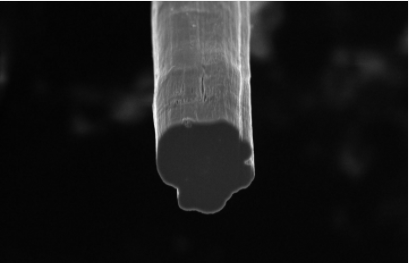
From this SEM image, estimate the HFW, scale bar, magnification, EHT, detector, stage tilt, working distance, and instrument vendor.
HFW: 42.2 µm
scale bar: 5000 nm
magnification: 4.908 K X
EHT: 5 kV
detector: TLD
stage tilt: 20°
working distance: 3.9 mm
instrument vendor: FEI